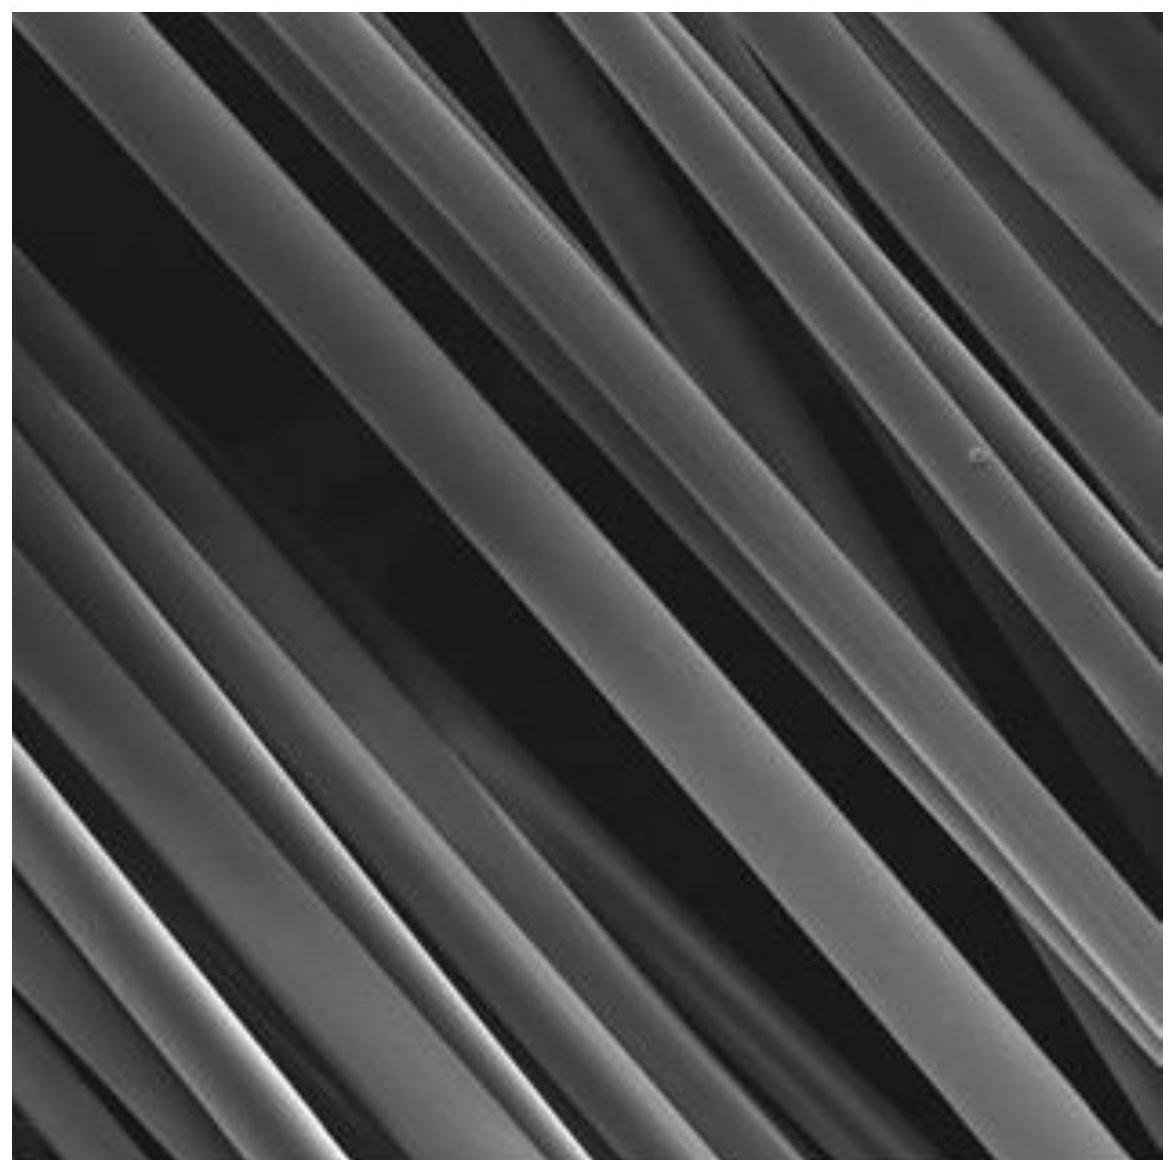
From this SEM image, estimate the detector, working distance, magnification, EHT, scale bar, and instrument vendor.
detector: SE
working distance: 4.98 mm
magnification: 2 K X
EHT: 5 kV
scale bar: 20000 nm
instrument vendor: Tescan